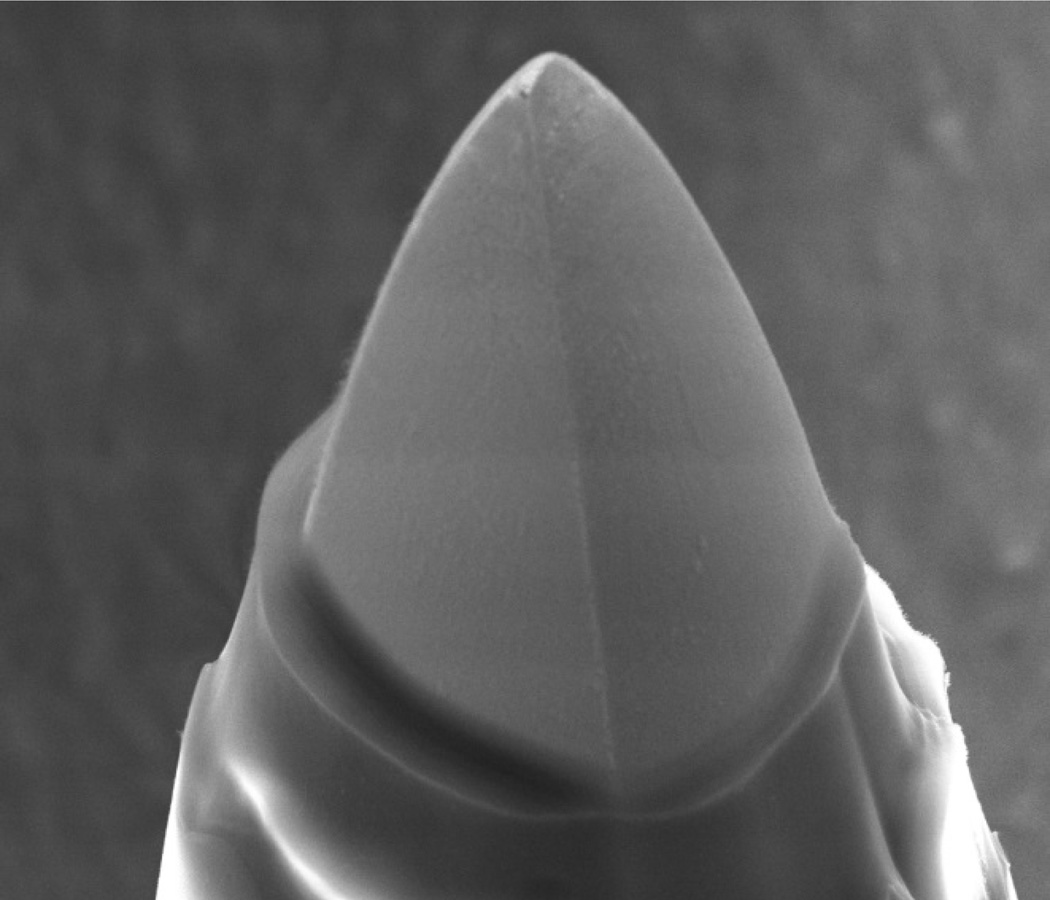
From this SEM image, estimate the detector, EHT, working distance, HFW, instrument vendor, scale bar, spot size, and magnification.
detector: ETD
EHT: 20 kV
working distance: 24.6 mm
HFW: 74.6 µm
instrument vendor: FEI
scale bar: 30000 nm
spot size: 5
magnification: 2 K X